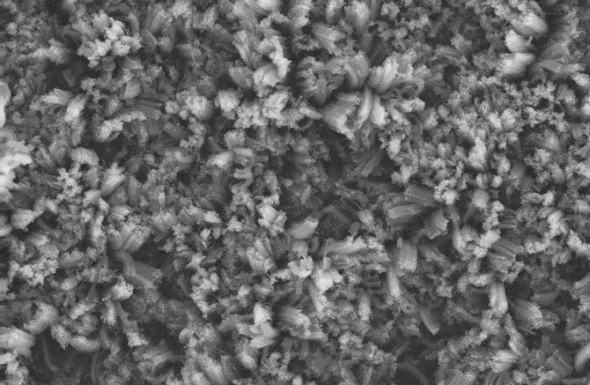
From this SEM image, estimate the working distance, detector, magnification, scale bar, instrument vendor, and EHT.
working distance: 6 mm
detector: SE2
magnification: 0.5 K X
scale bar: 20000 nm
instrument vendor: Zeiss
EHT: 10 kV